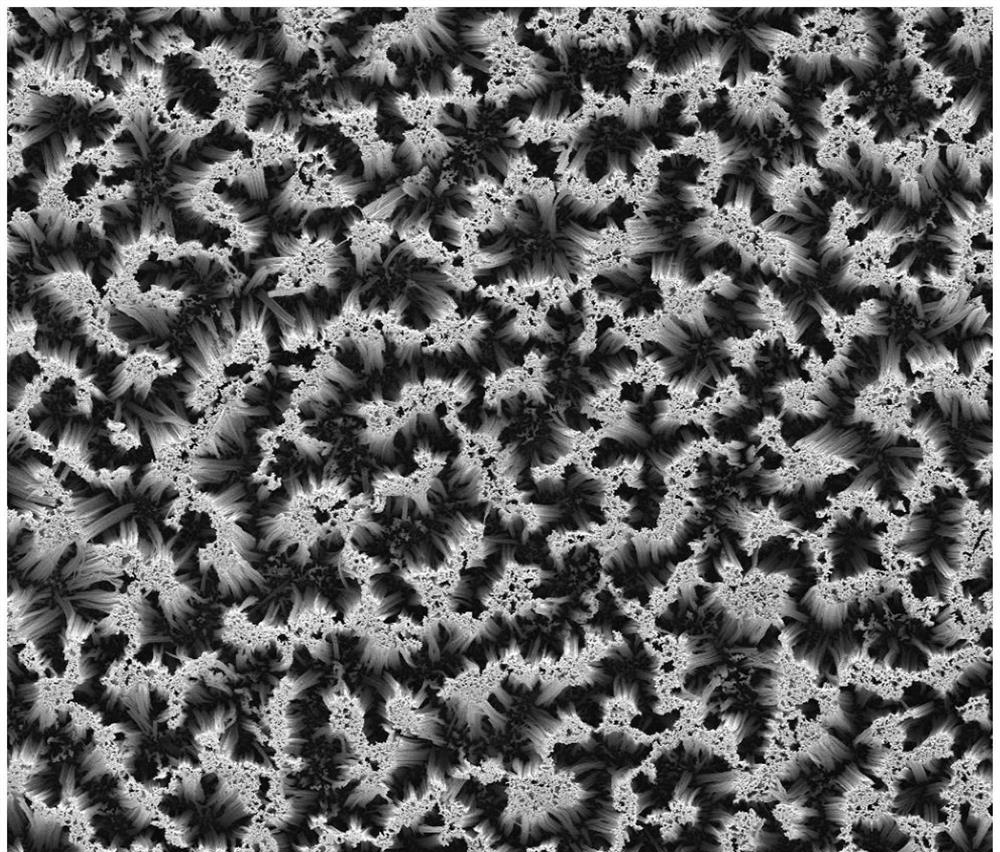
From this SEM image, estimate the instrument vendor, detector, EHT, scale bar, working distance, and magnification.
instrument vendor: FEI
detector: ETD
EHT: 10 kV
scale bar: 10000 nm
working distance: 10.3 mm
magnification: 10 K X